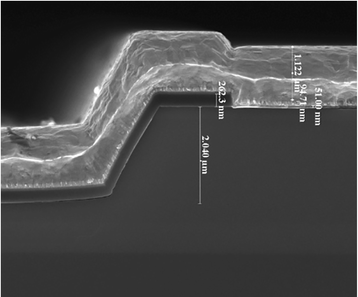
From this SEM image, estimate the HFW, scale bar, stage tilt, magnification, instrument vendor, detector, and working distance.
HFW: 7.46 µm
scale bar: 3000 nm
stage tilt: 0°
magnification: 40 K X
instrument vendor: FEI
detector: TLD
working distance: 4.9 mm